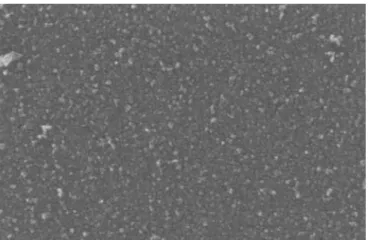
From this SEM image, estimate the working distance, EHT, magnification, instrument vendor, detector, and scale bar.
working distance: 5 mm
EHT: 5 kV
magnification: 100 K X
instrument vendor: Zeiss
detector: InLens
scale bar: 100 nm